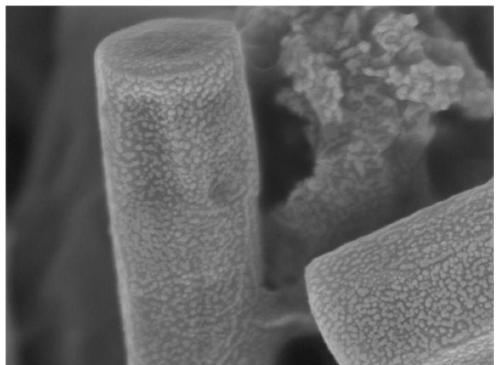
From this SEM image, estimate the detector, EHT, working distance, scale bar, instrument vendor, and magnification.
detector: InBeam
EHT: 5 kV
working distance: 5.95 mm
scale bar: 500 nm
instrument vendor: Tescan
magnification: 100 K X